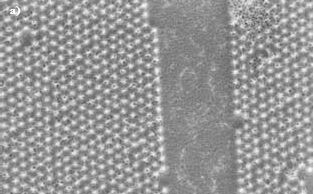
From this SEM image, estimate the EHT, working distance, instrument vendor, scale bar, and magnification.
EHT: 2 kV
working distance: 5 mm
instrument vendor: Zeiss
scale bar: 1000 nm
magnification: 25 K X